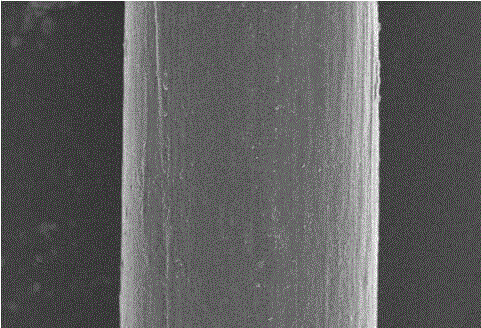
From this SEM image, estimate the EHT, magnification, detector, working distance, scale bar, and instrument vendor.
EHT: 5 kV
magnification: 1 K X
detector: SE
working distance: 7.6 mm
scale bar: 50000 nm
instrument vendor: Hitachi